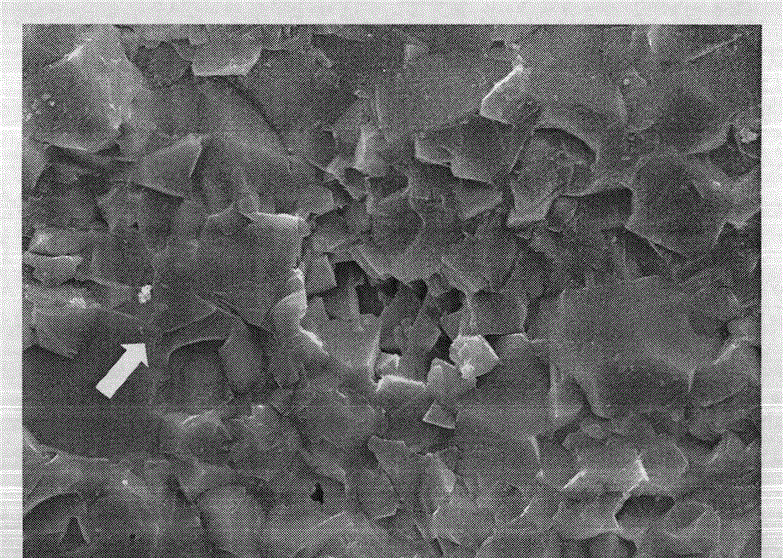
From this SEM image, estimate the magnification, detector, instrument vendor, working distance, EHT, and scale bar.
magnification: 2 K X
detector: SEI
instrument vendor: JEOL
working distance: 11.5 mm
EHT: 20 kV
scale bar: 10000 nm